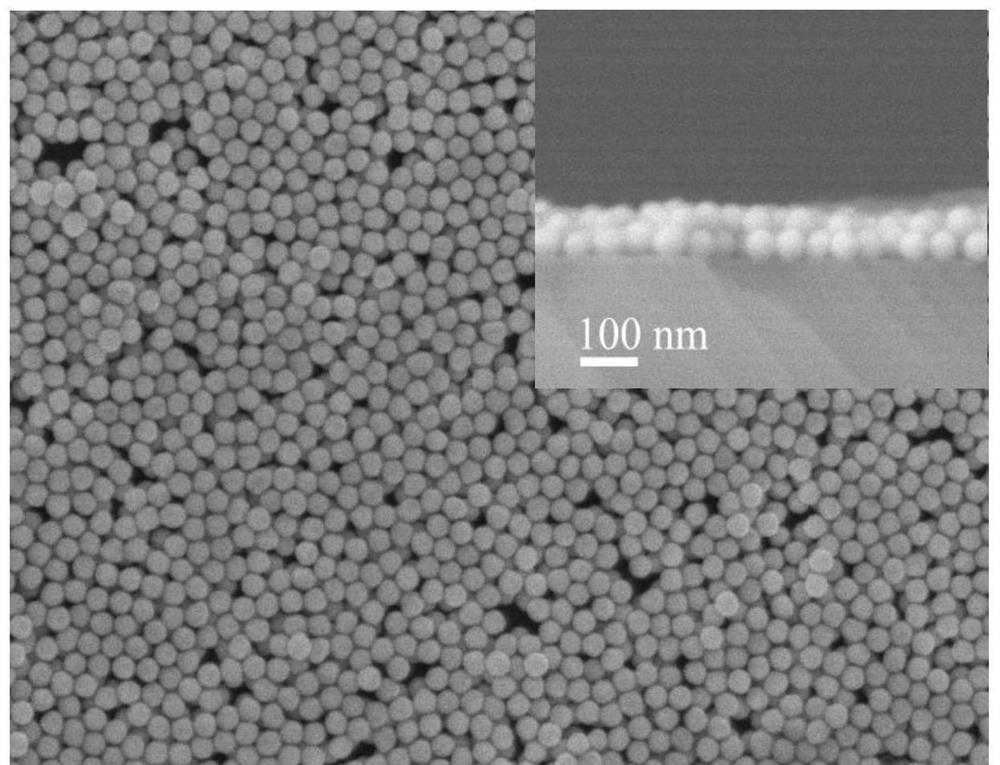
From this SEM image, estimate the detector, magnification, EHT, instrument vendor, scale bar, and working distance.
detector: SEI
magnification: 60 K X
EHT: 5 kV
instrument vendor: JEOL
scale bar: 100 nm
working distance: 6.2 mm